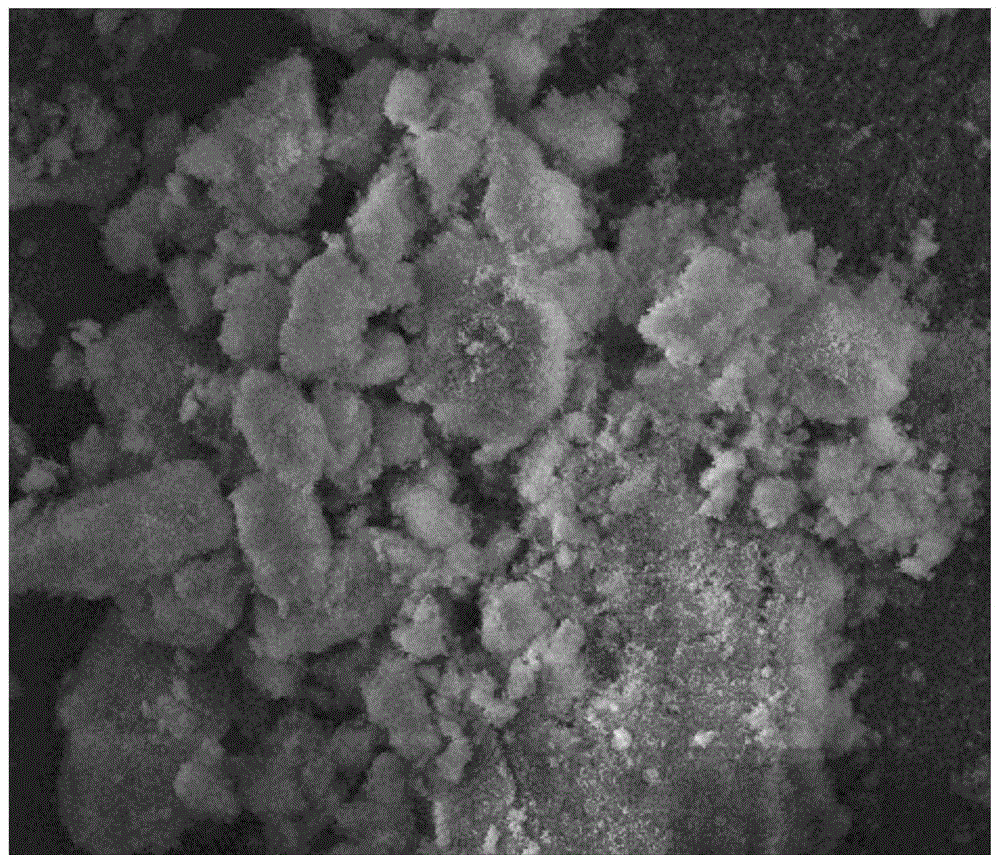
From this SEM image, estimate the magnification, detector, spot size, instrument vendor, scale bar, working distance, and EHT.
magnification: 2 K X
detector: ETD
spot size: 3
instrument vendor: FEI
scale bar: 50000 nm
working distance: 12 mm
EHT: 10 kV